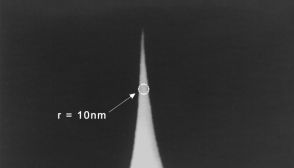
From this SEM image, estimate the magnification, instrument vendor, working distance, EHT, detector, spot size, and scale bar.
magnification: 100 K X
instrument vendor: FEI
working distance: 2.1 mm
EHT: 5 kV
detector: SE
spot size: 3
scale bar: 180 nm